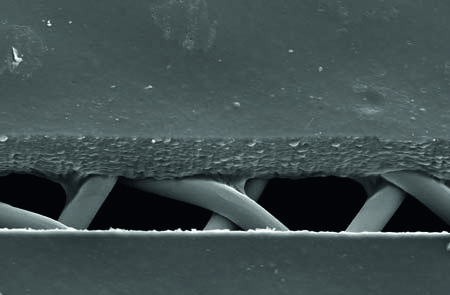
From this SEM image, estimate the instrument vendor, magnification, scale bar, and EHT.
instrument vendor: JEOL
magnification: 0.5 K X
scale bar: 50000 nm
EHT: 17 kV